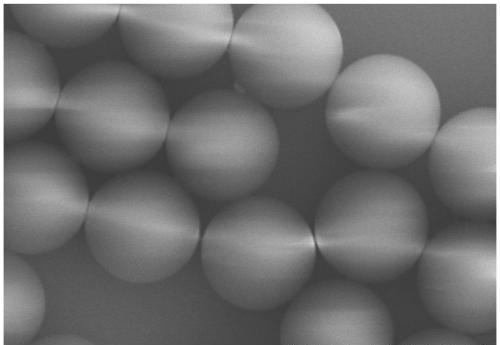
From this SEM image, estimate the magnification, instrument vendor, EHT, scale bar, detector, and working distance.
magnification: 10 K X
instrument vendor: Hitachi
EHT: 10 kV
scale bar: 5000 nm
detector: SE(UL)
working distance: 13.6 mm